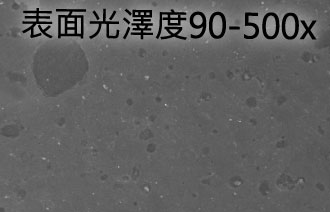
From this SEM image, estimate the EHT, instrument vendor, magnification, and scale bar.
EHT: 20 kV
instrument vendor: JEOL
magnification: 0.5 K X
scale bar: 20000 nm